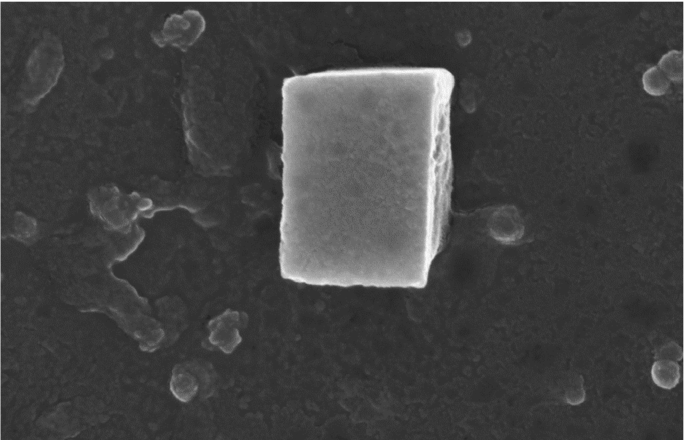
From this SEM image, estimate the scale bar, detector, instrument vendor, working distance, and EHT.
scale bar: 300 nm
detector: InLens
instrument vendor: Zeiss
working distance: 7.7 mm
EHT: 15 kV